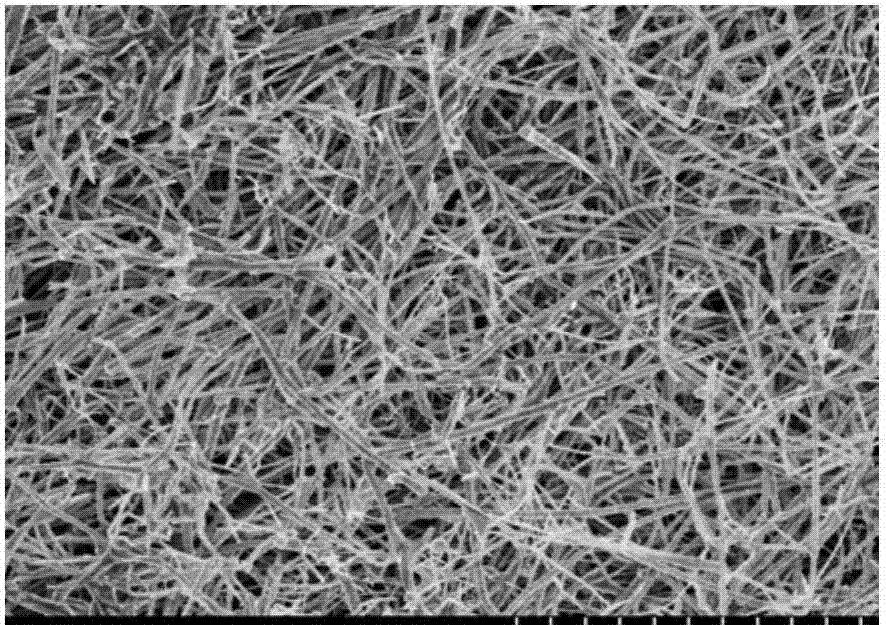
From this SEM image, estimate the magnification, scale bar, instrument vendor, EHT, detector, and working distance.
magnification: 5 K X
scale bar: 10000 nm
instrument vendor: Hitachi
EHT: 5 kV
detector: SE(M,LA0)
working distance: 9 mm